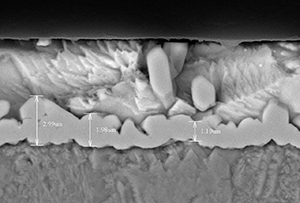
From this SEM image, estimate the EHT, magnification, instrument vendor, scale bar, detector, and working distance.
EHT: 15 kV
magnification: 7 K X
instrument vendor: Hitachi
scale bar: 5000 nm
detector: BSE3D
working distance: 8.5 mm